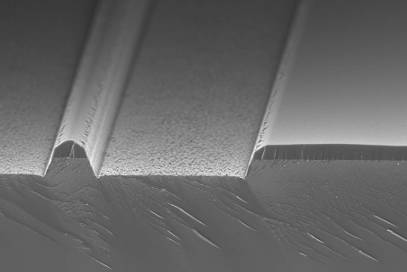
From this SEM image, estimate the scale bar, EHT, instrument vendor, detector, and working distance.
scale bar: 3000 nm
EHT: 5 kV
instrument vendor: Zeiss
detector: InLens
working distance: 2 mm